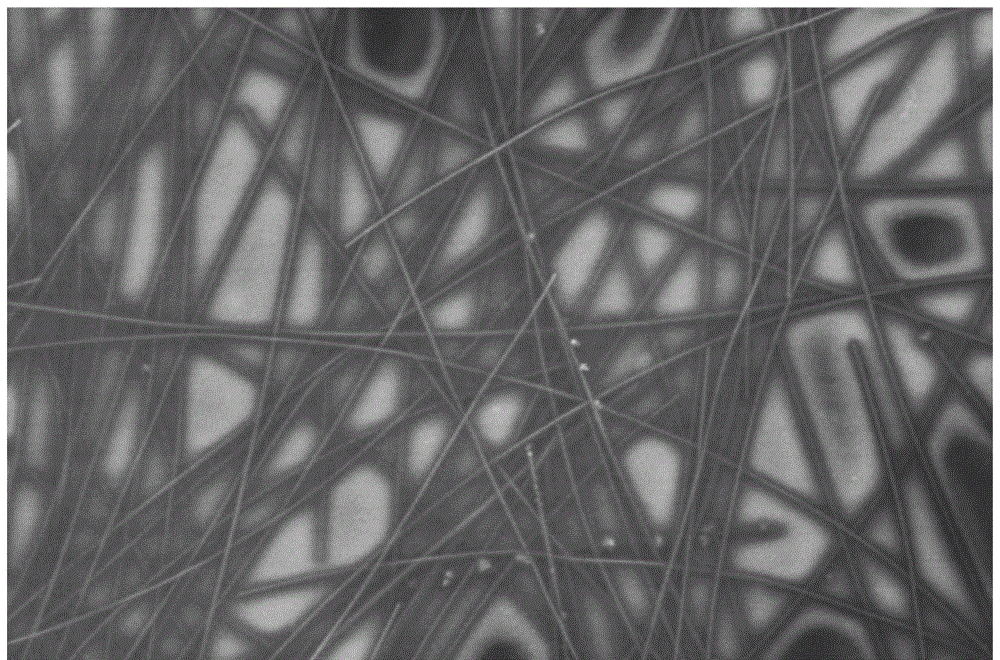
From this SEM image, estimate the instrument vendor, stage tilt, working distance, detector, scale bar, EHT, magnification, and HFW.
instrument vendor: FEI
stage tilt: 0°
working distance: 4.2 mm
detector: TLD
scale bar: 2000 nm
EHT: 2 kV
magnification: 60 K X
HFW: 6.91 µm